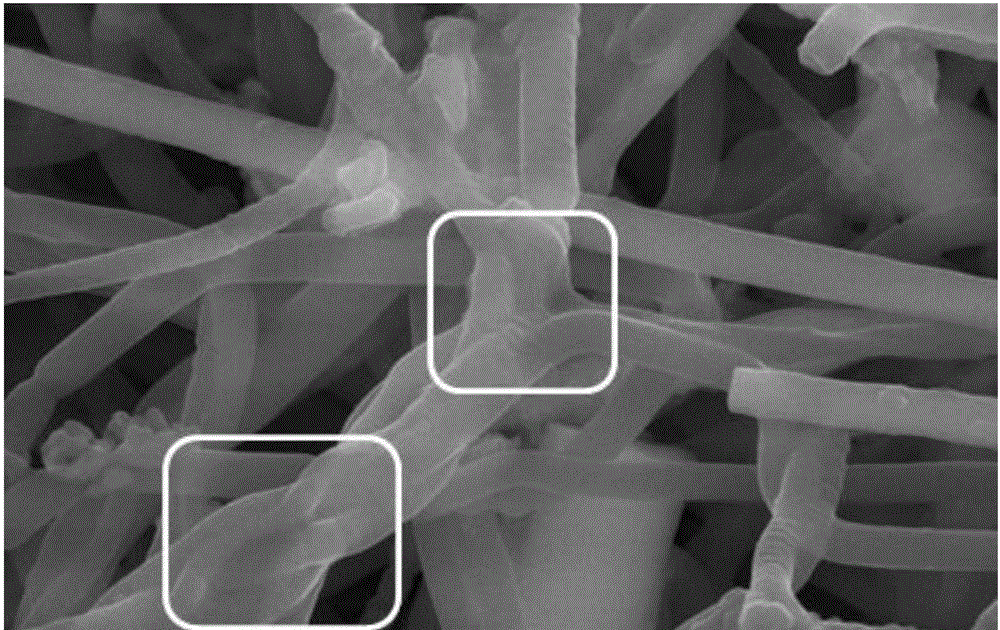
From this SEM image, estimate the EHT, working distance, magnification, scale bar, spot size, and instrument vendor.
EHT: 20 kV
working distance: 6.7 mm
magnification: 80 K X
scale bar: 2000 nm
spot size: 3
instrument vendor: FEI